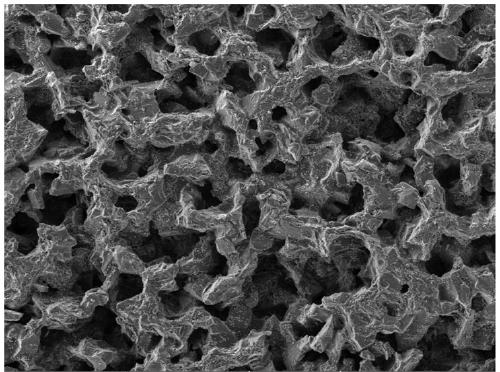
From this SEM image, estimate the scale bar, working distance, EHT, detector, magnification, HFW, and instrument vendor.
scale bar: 200000 nm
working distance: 11.45 mm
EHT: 15 kV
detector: SE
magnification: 0.2 K X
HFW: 1080 µm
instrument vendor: Tescan